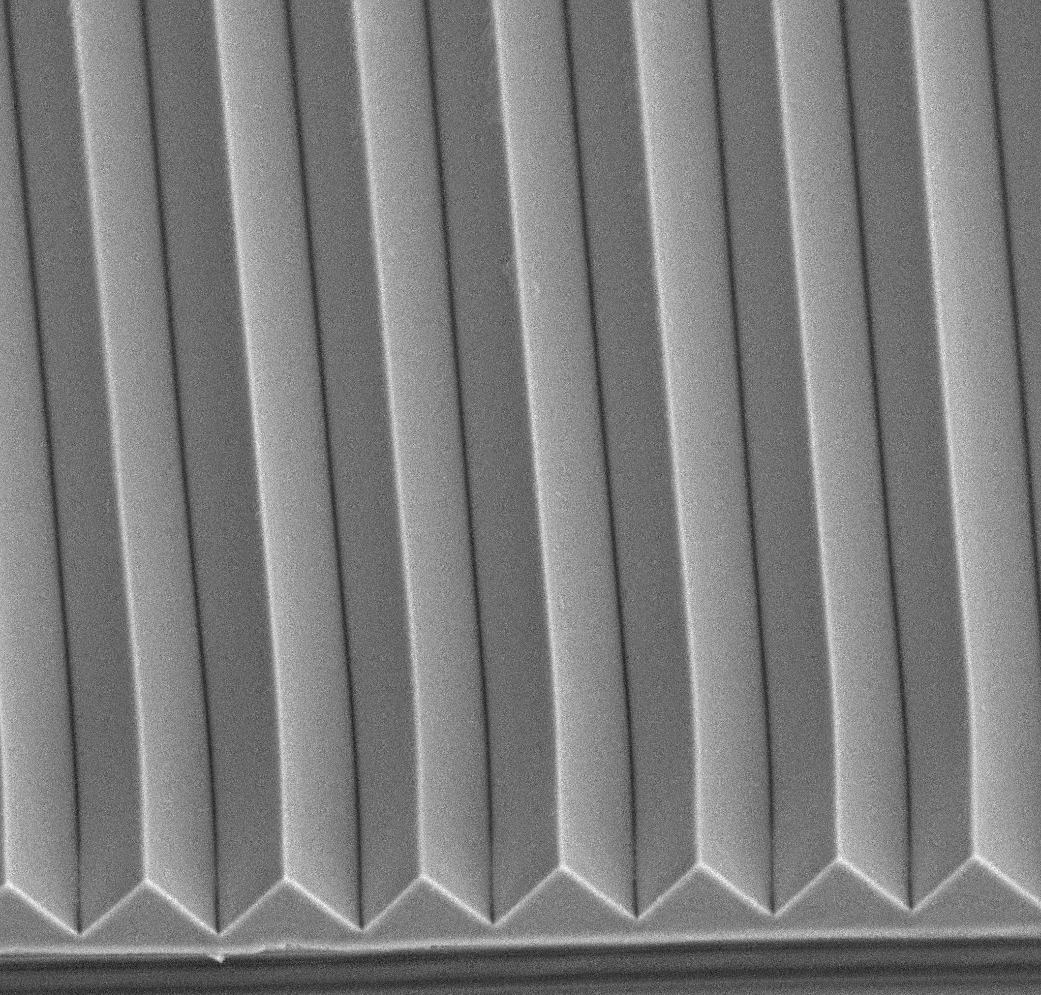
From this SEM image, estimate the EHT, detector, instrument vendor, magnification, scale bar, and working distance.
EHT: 15 kV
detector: Mix 50%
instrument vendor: other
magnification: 1.5 K X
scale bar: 20000 nm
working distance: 8.1 mm